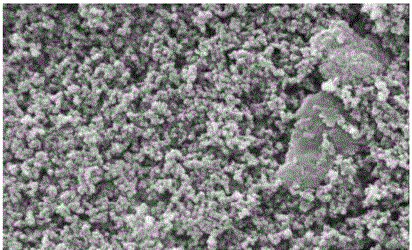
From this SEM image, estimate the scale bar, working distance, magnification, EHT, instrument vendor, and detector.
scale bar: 200 nm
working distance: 10.5 mm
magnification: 50 K X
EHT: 20 kV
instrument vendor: Zeiss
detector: SE1